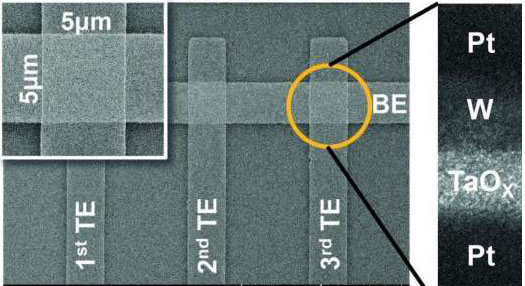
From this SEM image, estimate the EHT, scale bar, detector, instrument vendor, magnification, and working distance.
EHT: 10 kV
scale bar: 20000 nm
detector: SE(UL)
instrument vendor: Hitachi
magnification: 2.5 K X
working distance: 7 mm